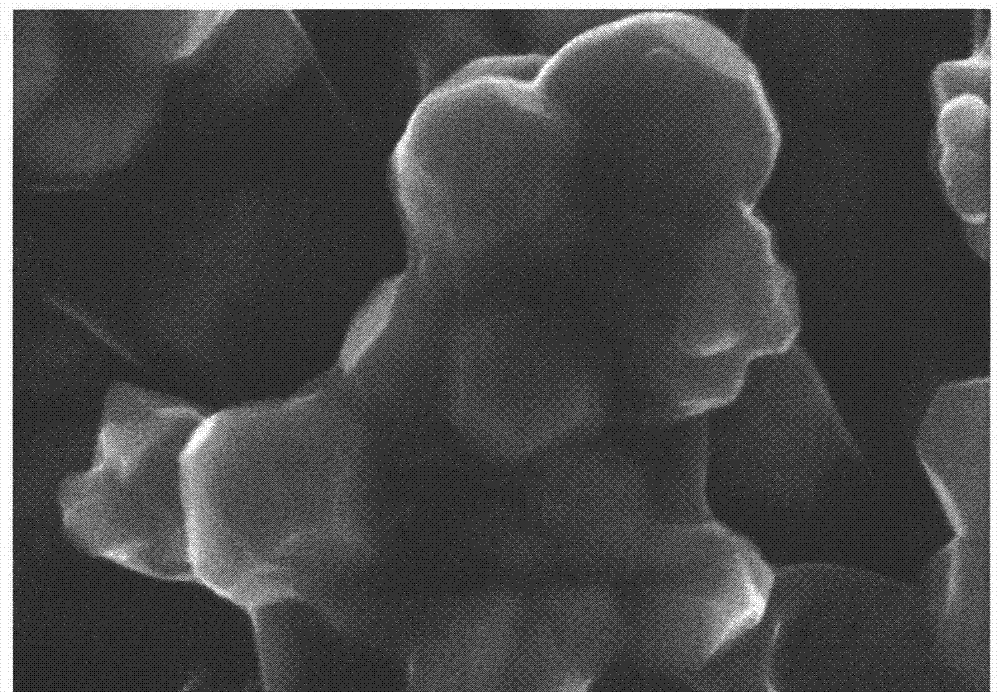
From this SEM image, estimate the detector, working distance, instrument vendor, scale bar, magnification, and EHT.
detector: InLens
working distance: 6.5 mm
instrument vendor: Zeiss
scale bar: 200 nm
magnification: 100 K X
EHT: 5 kV